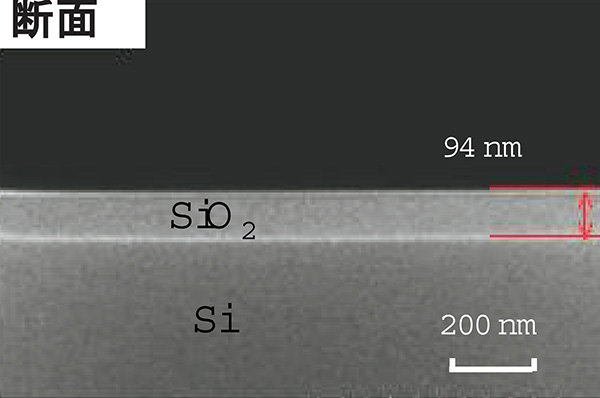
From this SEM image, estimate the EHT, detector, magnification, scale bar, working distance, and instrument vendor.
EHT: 10 kV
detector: SE(U)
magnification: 100 K X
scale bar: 500 nm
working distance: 12.9 mm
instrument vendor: Hitachi